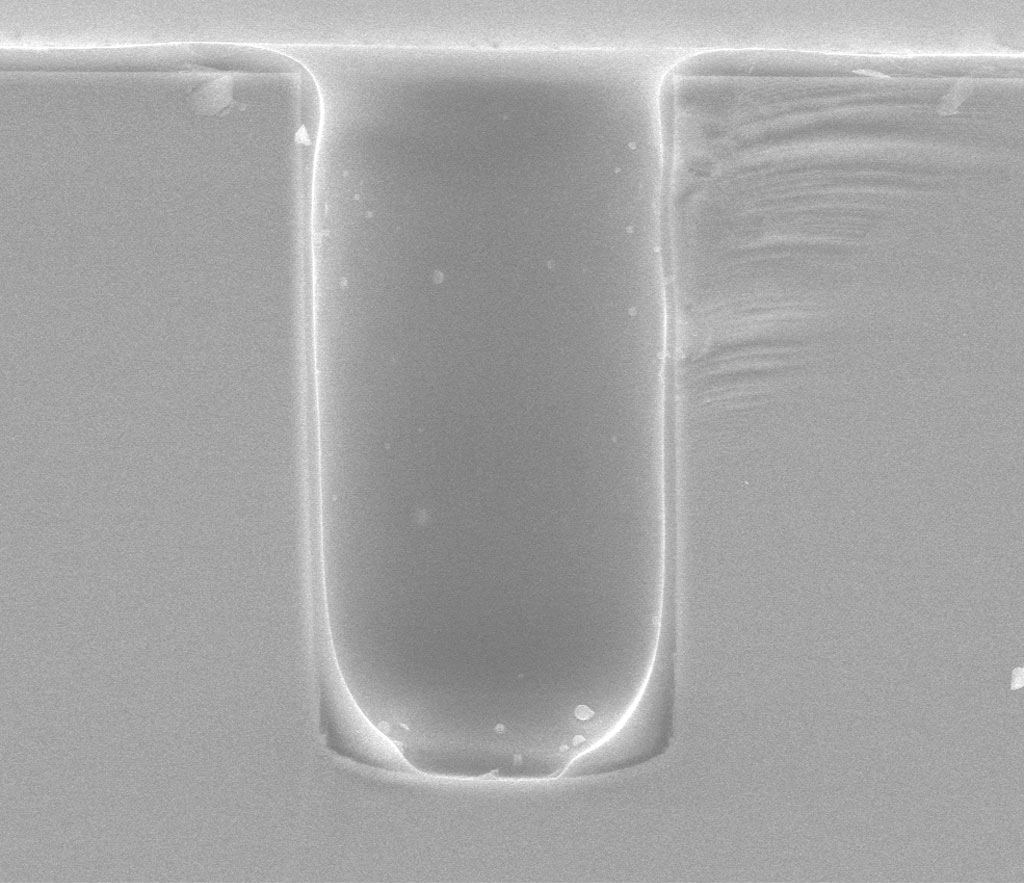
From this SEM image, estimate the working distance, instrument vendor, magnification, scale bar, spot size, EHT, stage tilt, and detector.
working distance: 6 mm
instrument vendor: FEI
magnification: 1 K X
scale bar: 100000 nm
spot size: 2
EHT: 30 kV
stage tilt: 0°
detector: LFD